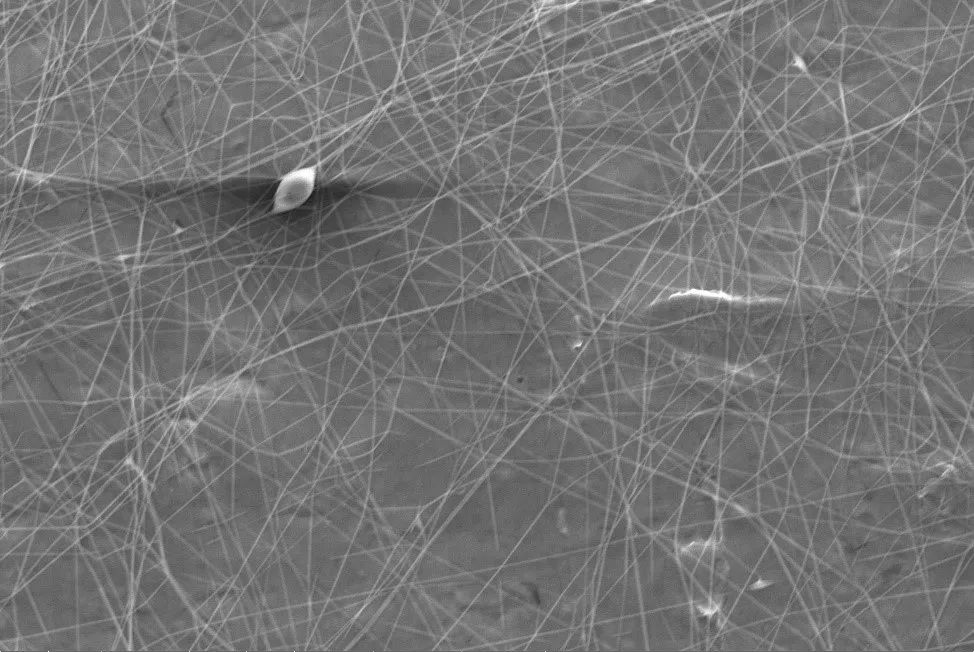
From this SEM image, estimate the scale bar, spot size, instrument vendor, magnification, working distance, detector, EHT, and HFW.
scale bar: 30000 nm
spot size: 3.5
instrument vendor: FEI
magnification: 1.6 K X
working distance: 21.1 mm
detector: ETD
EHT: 10 kV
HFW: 79.4 µm